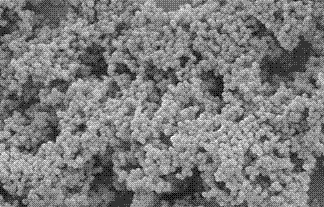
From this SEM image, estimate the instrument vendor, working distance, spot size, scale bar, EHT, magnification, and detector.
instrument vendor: FEI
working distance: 6.9 mm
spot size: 3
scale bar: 2000 nm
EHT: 5 kV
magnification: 20 K X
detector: SE